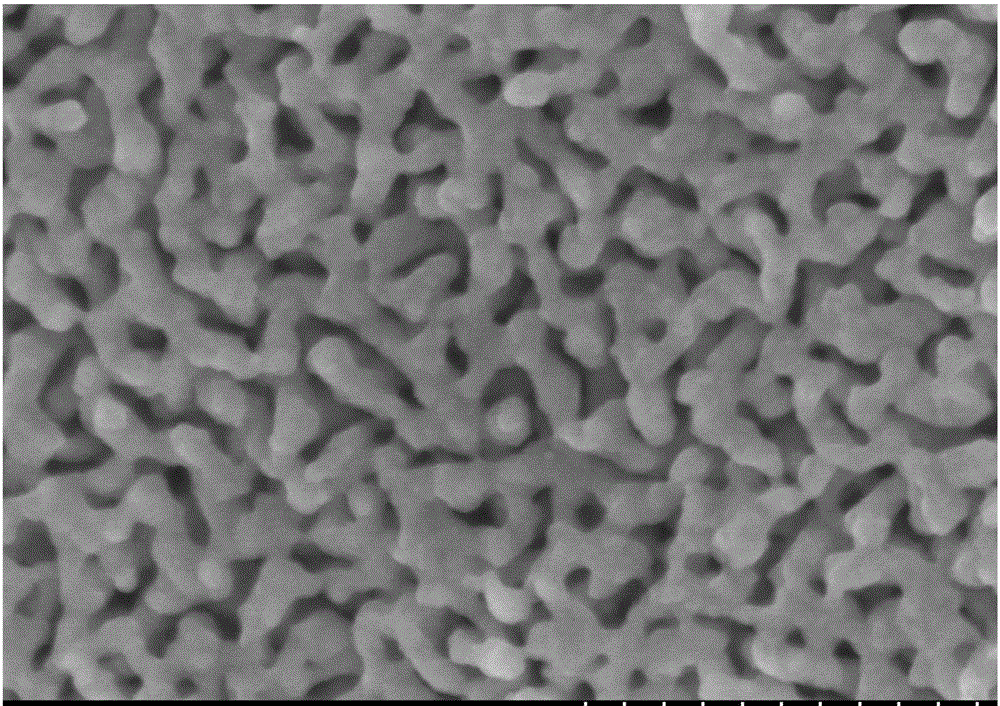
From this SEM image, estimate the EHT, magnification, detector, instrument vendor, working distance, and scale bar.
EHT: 5 kV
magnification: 100 K X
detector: SE(M)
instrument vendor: Hitachi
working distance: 8.1 mm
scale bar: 500 nm